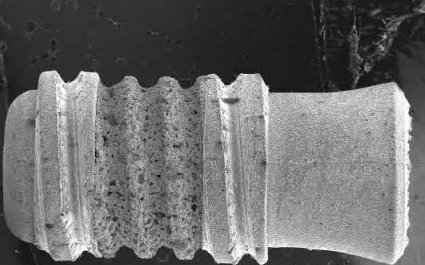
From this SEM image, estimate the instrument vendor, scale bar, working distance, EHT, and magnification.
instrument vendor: JEOL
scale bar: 1000000 nm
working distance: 39 mm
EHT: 15 kV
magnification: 0.011 K X